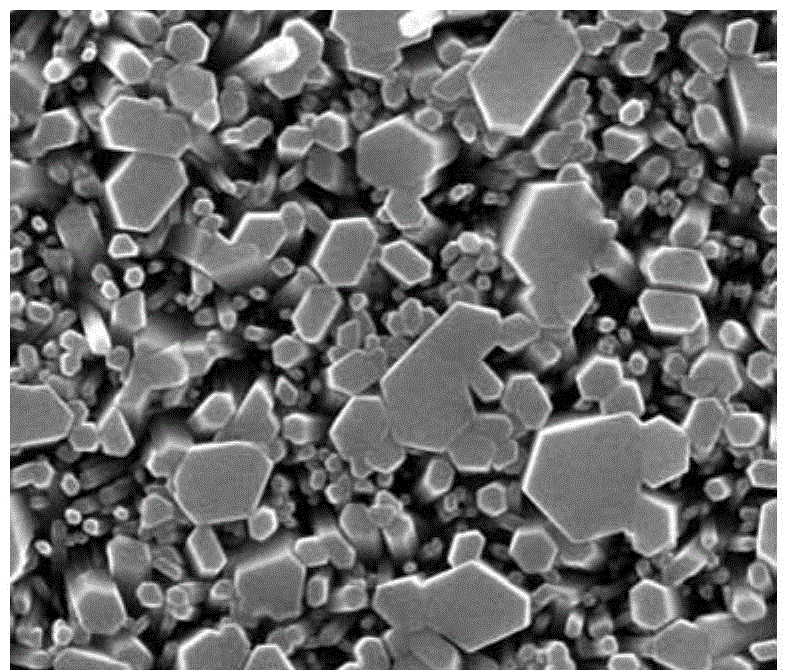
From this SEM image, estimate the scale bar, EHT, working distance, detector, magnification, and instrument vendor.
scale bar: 1000 nm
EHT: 30 kV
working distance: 10.5 mm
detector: SE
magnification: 100 K X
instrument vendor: FEI